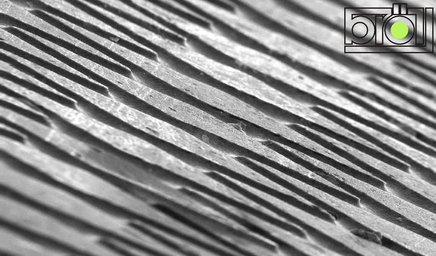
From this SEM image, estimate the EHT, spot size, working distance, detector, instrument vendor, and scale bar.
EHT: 24 kV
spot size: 320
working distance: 18 mm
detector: SE1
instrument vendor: Zeiss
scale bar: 20000 nm